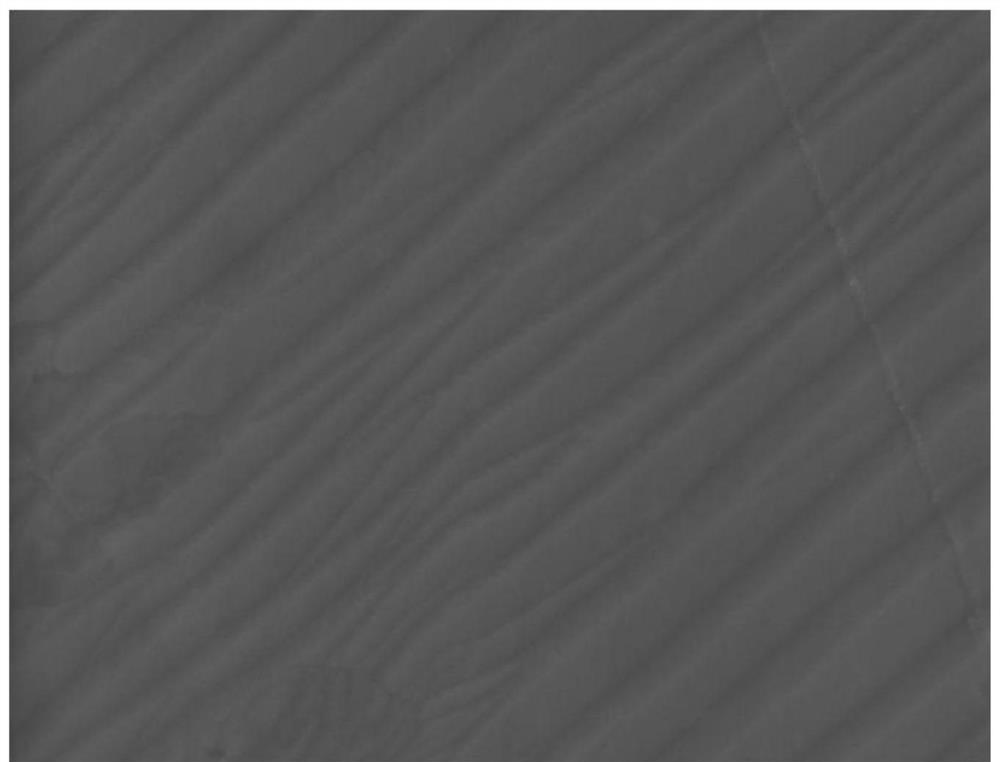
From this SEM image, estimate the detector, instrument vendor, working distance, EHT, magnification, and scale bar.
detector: SE(U)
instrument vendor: Hitachi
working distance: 8.2 mm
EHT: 5 kV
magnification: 50 K X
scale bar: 1000 nm